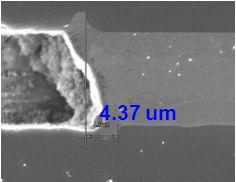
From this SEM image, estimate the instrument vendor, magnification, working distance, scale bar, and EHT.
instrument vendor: Hitachi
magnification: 4 K X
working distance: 5.7 mm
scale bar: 10000 nm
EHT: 10 kV